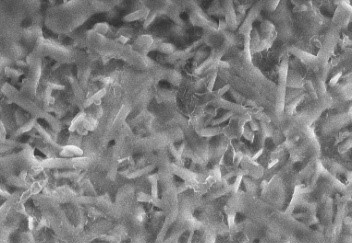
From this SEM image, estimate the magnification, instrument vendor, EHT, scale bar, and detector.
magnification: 1 K X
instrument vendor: Hitachi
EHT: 30 kV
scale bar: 50000 nm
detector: SE